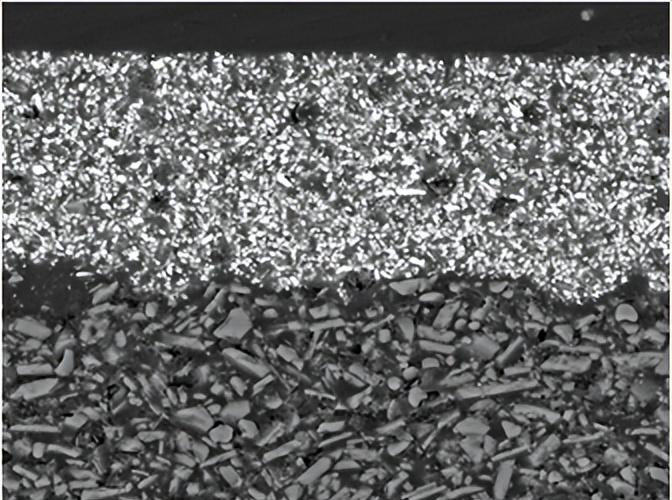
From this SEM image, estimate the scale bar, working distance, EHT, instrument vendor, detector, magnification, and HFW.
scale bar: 20000 nm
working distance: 14.84 mm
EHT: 20 kV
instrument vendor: Tescan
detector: BSE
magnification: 2.29 K X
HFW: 121 µm